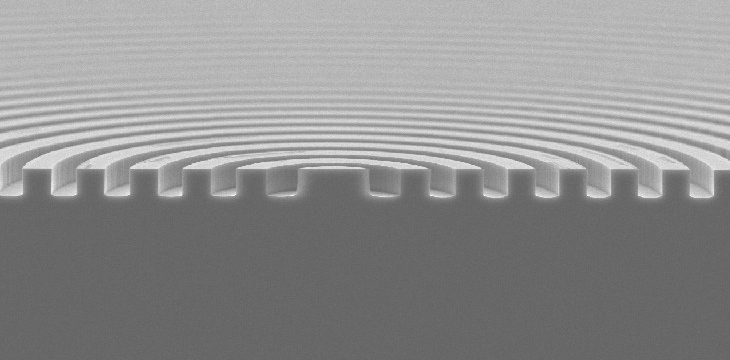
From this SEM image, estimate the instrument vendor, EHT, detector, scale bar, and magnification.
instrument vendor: JEOL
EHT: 15 kV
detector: SEI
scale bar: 10000 nm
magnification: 1.8 K X